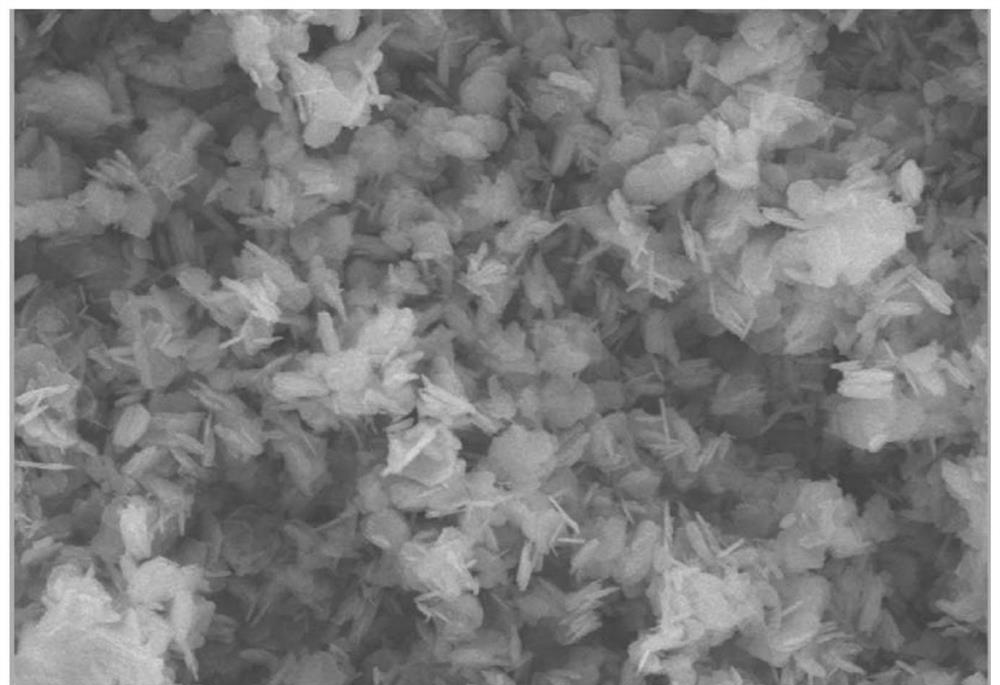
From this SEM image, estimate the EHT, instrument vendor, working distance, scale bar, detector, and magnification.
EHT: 10 kV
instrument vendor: JEOL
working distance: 10 mm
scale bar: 1000 nm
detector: SEI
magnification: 8 K X